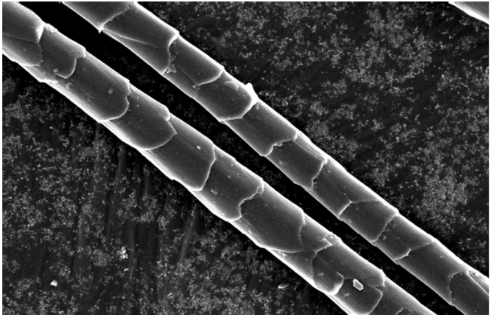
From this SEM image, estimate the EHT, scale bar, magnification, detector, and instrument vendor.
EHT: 10 kV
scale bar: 10000 nm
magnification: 1 K X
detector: SEI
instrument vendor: JEOL